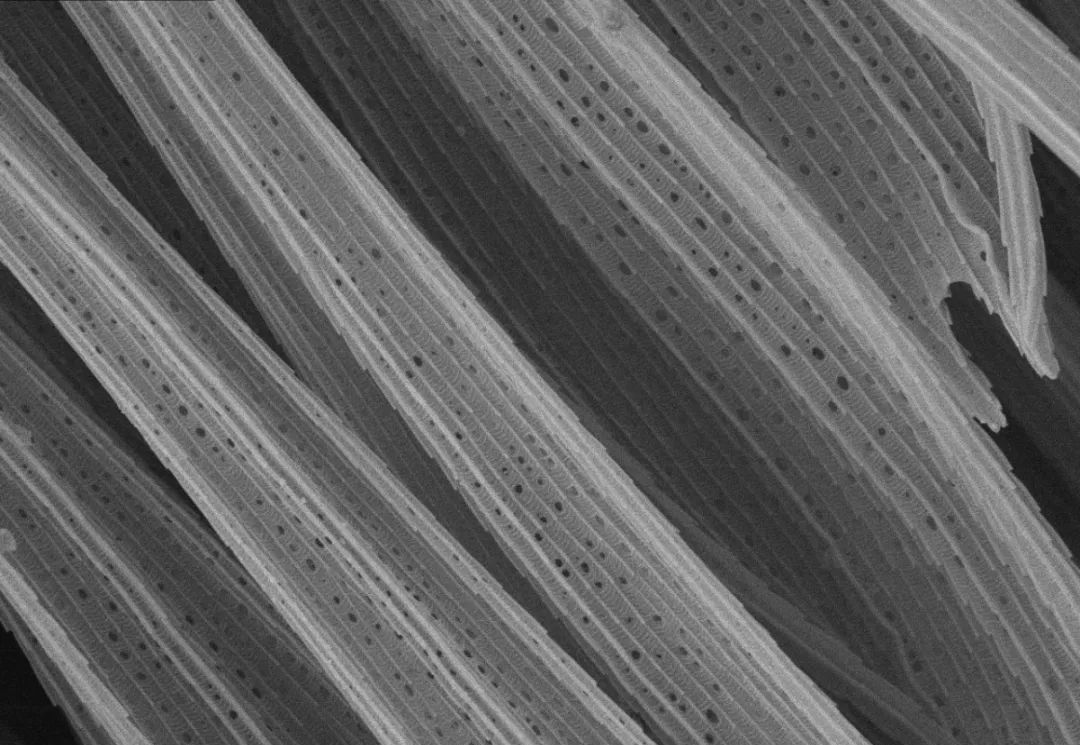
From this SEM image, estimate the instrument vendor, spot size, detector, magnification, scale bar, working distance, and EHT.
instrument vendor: other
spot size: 13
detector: SEI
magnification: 5 K X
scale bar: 10000 nm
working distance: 5.1 mm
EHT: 7 kV